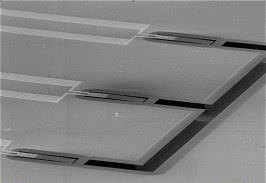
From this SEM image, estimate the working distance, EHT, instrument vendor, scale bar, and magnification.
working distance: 48 mm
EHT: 26 kV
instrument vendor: other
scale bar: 400000 nm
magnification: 0.045 K X